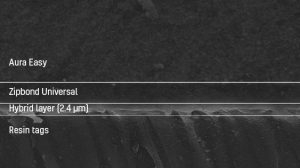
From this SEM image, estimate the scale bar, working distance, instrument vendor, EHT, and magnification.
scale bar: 10000 nm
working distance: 20 mm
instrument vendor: JEOL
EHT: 15 kV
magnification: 2 K X